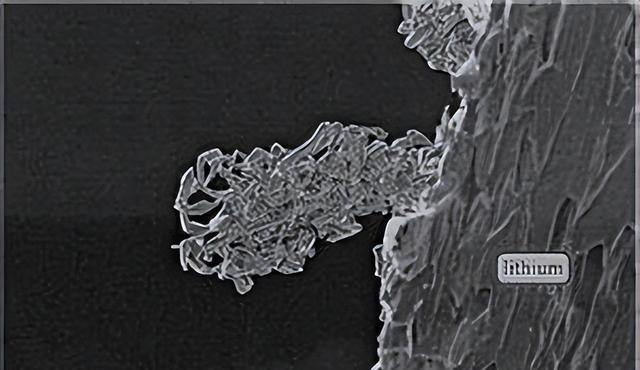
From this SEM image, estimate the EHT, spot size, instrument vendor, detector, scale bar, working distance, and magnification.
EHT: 2 kV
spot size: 3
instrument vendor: FEI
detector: SE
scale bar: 20000 nm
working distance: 8.3 mm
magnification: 1.047 K X